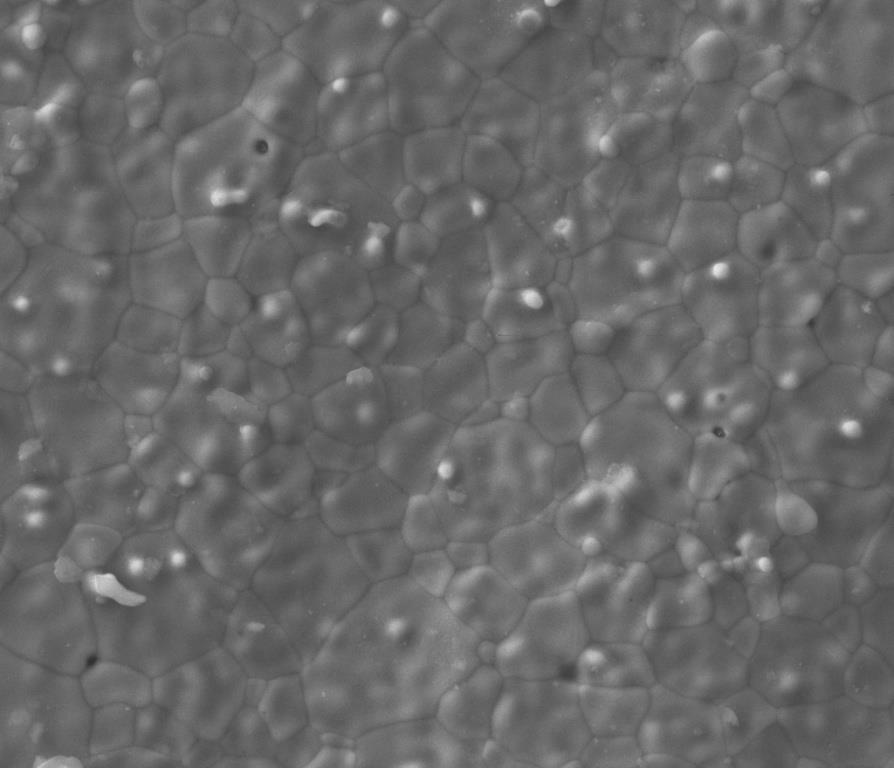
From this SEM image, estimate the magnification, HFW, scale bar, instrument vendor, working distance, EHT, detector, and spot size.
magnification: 5 K X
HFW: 59.7 µm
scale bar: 20000 nm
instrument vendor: FEI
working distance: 8.7 mm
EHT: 18 kV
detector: ETD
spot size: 4.5